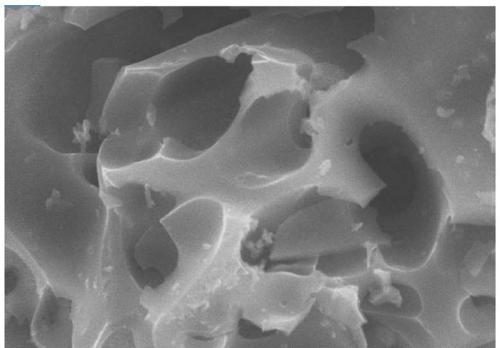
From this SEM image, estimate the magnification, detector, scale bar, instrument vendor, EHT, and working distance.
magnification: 10 K X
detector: SE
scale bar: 5000 nm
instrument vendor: Hitachi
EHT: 10 kV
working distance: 7.6 mm